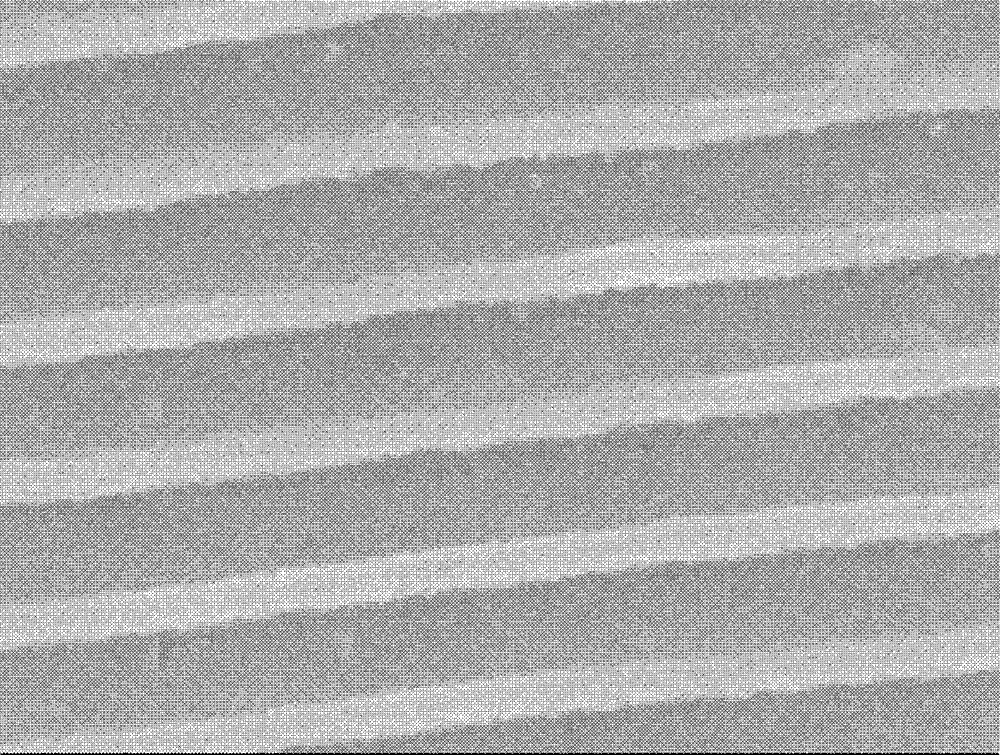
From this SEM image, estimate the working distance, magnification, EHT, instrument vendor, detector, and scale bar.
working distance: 6 mm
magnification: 25 K X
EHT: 8 kV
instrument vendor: JEOL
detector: SEI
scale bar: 1000 nm